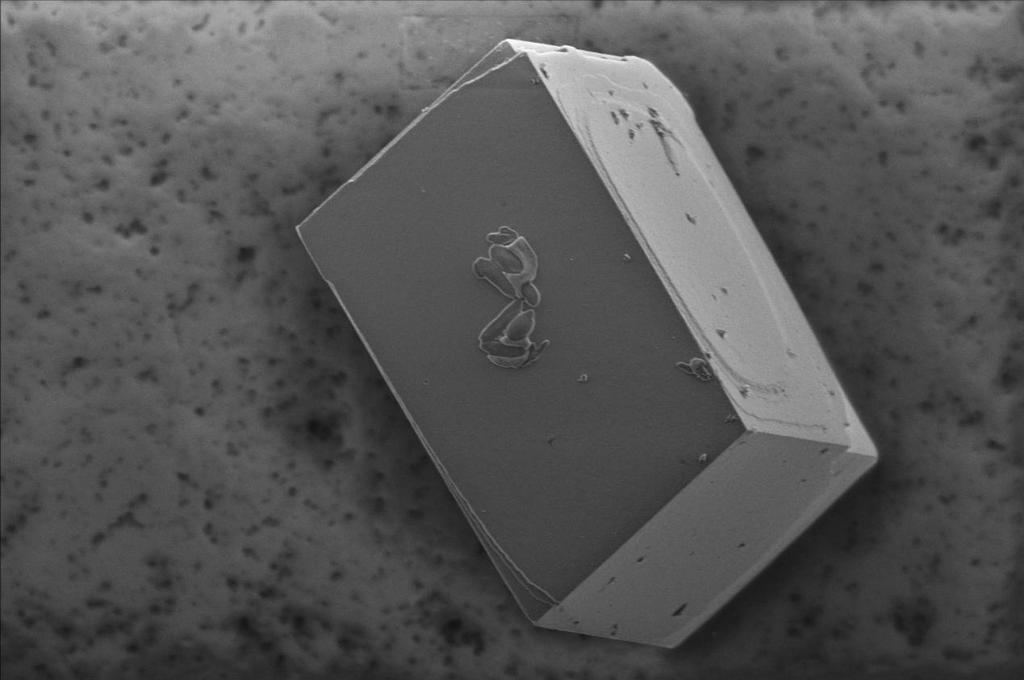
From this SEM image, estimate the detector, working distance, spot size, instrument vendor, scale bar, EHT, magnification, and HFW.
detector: ETD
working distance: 10.9 mm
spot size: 2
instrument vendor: FEI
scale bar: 20000 nm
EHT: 2 kV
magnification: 4.429 K X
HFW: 93.6 µm